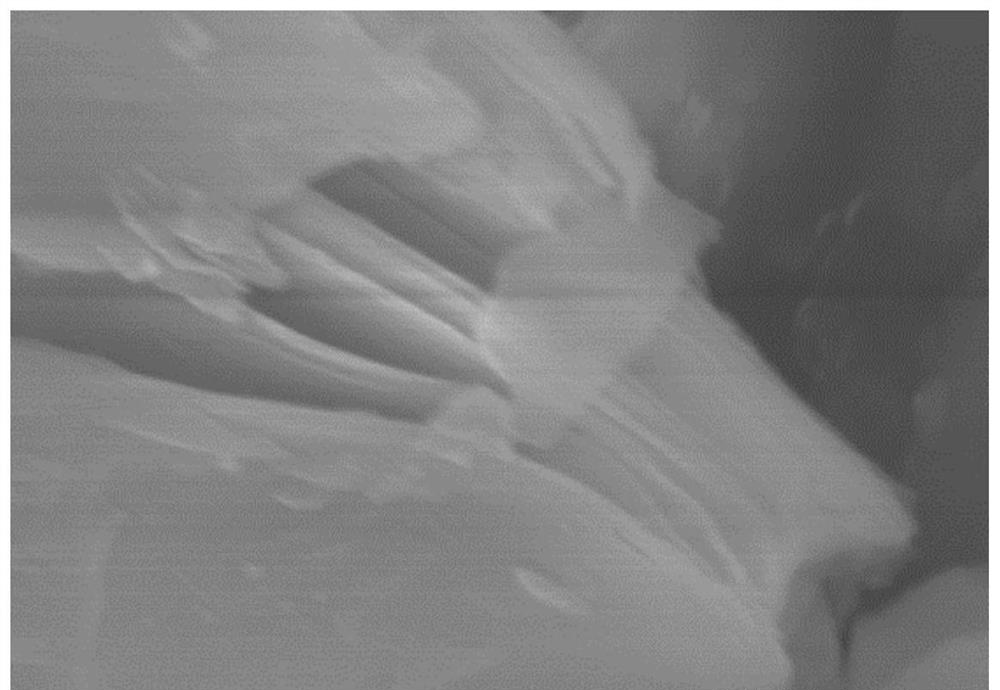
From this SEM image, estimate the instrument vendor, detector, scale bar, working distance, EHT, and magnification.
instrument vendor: Hitachi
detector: SE(M)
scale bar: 1000 nm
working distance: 10.3 mm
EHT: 10 kV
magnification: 40 K X